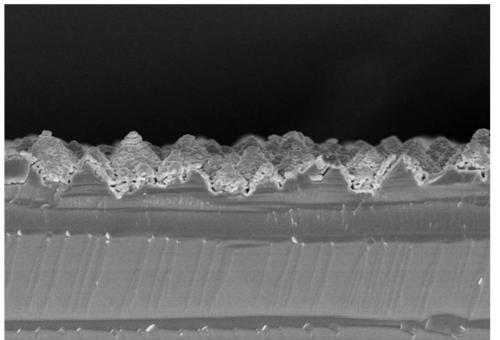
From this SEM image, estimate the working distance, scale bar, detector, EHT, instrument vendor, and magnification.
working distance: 11.4 mm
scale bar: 10000 nm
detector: SE(U)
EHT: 5 kV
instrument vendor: Hitachi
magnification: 5 K X